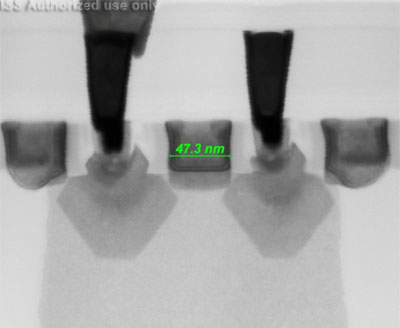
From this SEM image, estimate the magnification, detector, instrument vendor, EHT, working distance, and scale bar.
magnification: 800 K X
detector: STEM II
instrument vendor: FEI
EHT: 30 kV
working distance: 4.3 mm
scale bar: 100 nm